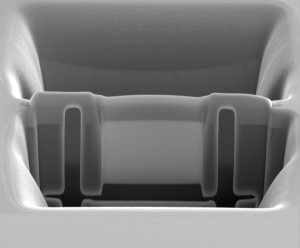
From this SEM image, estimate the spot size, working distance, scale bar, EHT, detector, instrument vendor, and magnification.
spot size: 4.5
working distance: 9.7 mm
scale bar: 5000 nm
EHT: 5 kV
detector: ETD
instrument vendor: FEI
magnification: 20 K X